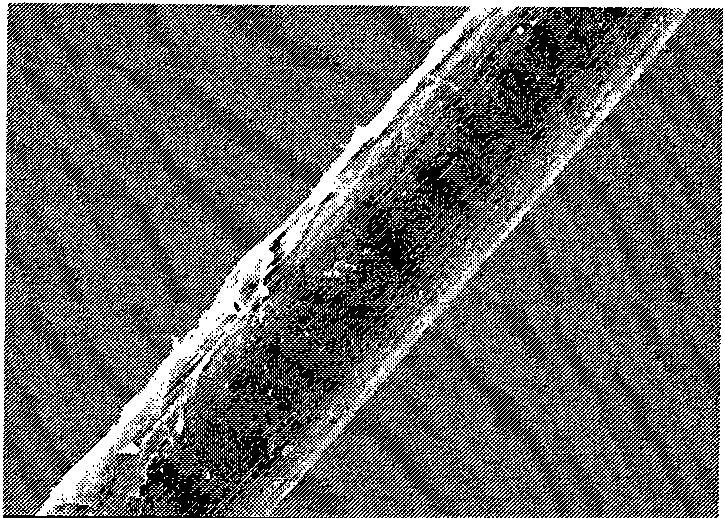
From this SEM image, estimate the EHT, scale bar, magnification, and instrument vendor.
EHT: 10 kV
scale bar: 10000 nm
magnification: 1 K X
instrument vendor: JEOL